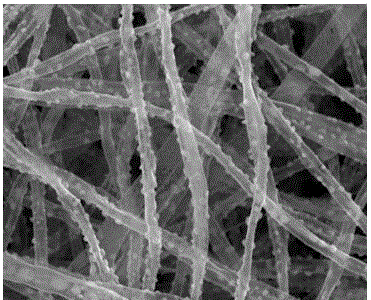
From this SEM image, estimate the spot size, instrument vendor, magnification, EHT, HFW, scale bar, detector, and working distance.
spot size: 2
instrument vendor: FEI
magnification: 50 K X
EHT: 10 kV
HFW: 2.98 µm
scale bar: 500 nm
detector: ETD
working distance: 4.7 mm